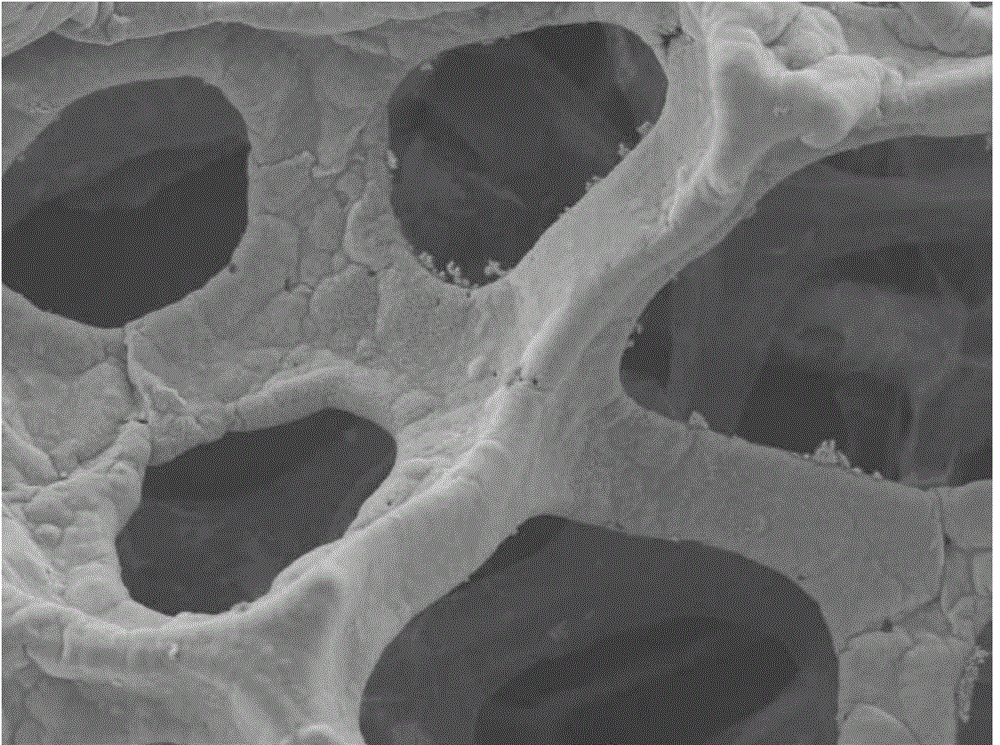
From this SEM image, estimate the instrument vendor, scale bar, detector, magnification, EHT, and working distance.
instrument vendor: JEOL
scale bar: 100000 nm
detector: SEI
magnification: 0.2 K X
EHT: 15 kV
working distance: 10.3 mm